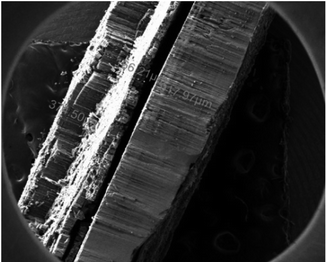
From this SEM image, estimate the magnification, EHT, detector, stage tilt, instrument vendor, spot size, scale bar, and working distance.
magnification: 0.07 K X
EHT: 10 kV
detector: ETD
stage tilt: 0°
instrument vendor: FEI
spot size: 3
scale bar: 1000000 nm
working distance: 8.8 mm